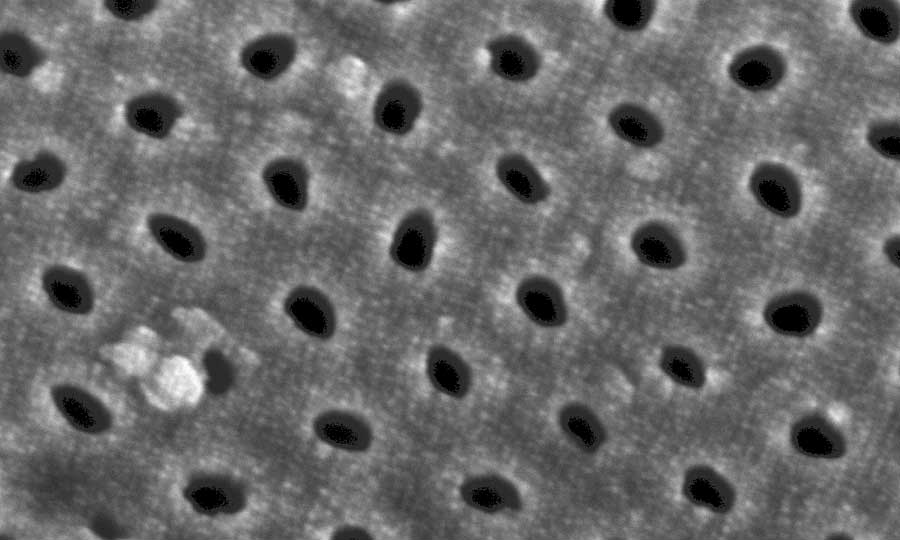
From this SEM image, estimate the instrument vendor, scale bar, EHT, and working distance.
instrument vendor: Zeiss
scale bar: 20 nm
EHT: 10 kV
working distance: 4 mm